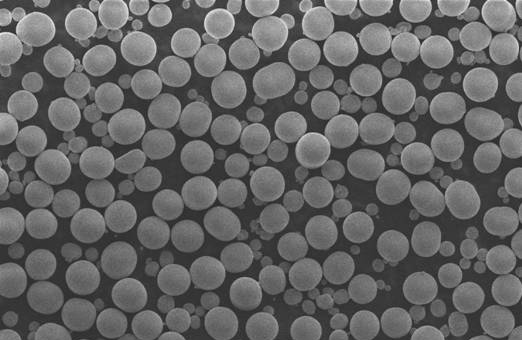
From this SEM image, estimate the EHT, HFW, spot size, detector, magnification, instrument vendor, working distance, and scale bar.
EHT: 6 kV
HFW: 207 µm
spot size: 6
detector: ETD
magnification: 1 K X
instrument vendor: Thermo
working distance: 11.6 mm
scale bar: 50000 nm